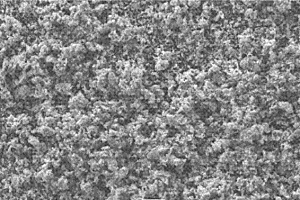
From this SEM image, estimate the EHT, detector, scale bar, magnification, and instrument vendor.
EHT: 15 kV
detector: SEI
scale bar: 10000 nm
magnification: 1 K X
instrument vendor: JEOL